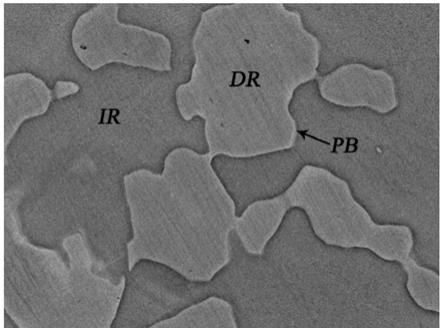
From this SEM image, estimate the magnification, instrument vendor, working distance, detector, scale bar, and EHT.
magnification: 5 K X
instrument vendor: JEOL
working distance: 12 mm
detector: COMPO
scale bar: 1000 nm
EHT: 15 kV